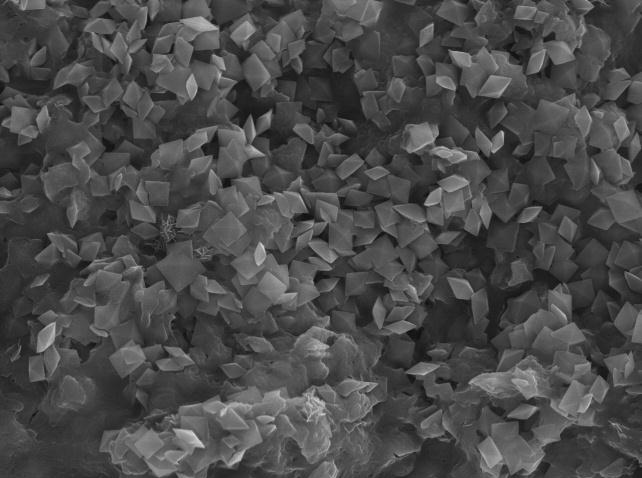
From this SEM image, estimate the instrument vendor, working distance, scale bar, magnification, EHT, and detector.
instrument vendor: JEOL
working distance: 15 mm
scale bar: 1000 nm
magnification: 3 K X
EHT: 20 kV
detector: SEI